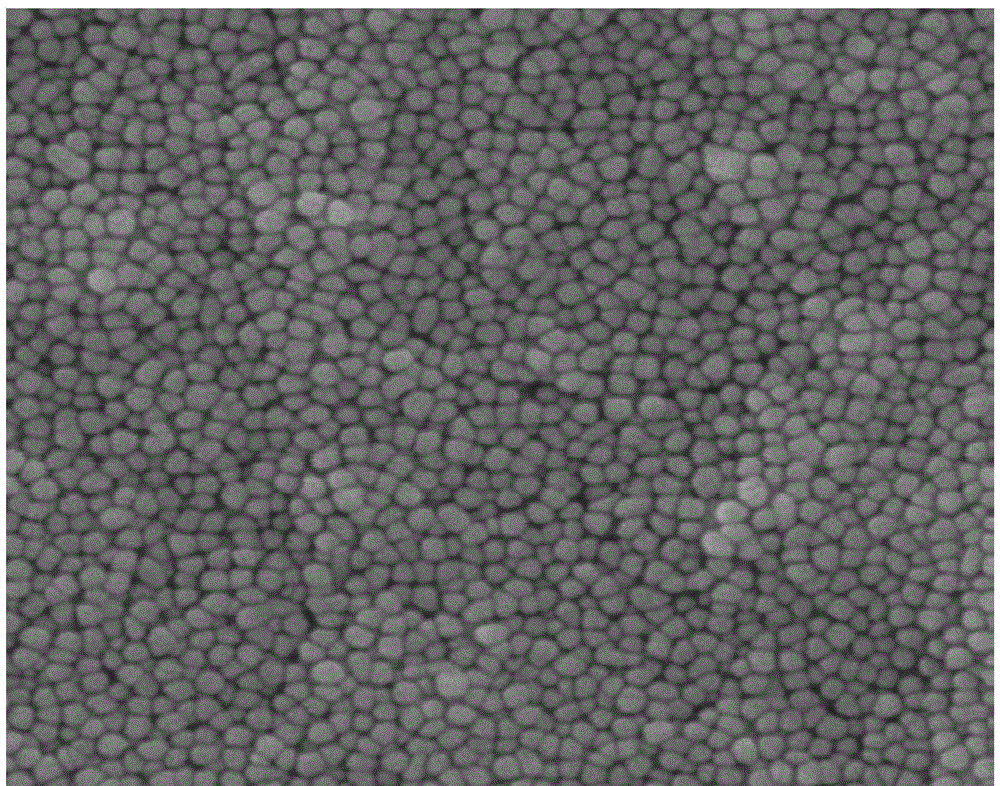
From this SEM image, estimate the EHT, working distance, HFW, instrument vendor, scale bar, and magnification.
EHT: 18 kV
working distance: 5.51 mm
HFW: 5.12 µm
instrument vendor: Tescan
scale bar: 1000 nm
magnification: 34.8 K X